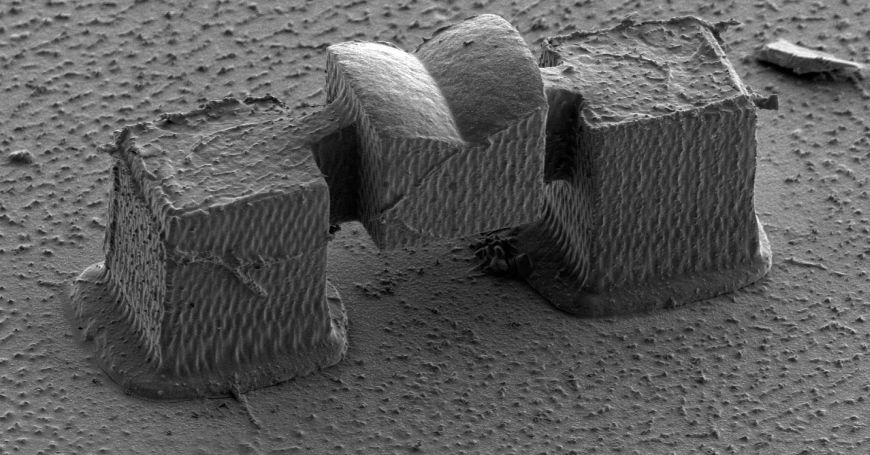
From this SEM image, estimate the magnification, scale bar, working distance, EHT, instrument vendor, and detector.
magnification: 1.5 K X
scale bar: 30000 nm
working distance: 16.7 mm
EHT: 1.5 kV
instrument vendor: Hitachi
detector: SE(L)